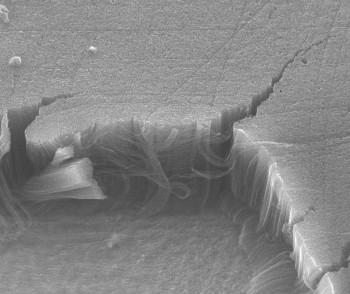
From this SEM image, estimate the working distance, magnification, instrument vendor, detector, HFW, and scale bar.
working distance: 11.8 mm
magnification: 4.294 K X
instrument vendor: FEI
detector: ETD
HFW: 62.98 µm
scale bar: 20000 nm